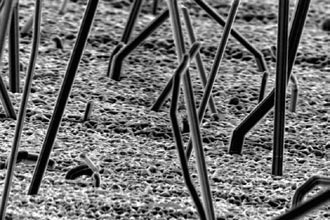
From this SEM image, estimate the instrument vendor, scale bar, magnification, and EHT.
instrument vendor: JEOL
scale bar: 10000 nm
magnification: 1 K X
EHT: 20 kV